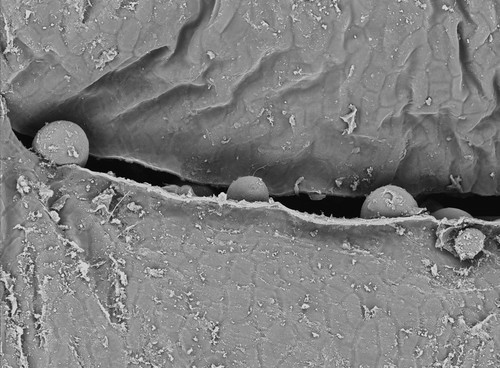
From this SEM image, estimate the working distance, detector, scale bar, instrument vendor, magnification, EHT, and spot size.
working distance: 8.4 mm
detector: BSE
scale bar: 100000 nm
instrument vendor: FEI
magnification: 0.245 K X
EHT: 15 kV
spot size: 3